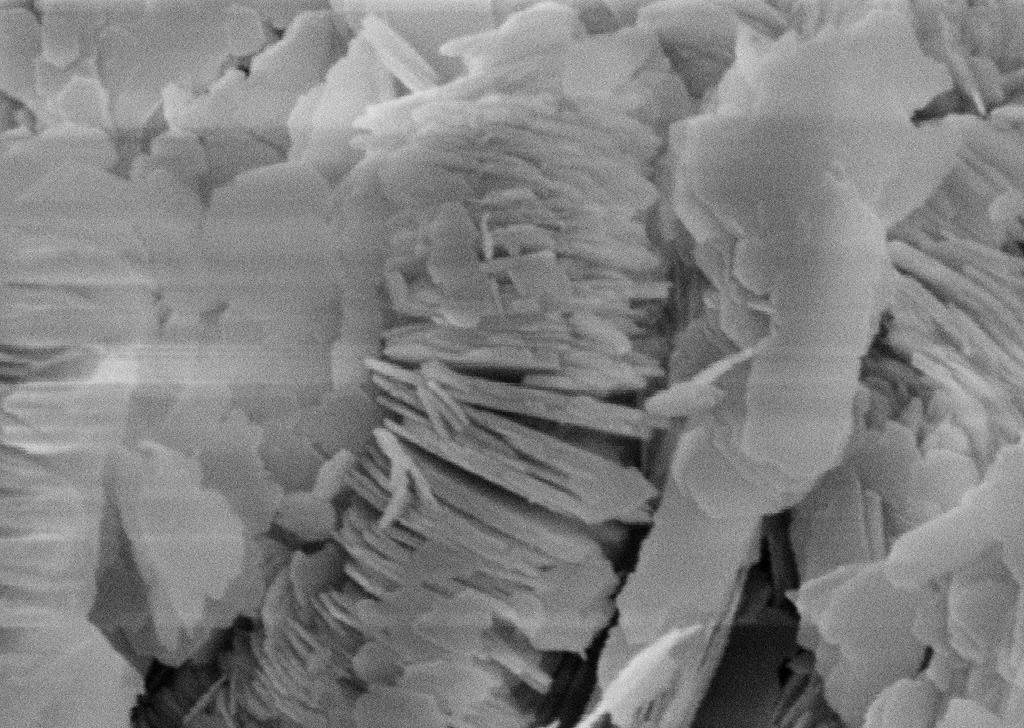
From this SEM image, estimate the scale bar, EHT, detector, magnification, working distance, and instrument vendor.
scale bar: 2000 nm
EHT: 10 kV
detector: SE1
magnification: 20 K X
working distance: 15 mm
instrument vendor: Zeiss